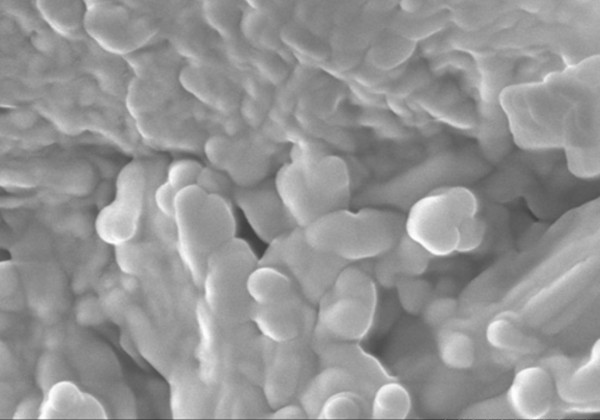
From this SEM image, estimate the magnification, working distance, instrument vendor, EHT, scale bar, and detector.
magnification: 30 K X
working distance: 12.9 mm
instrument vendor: Hitachi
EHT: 10 kV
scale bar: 1000 nm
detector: SE(U)